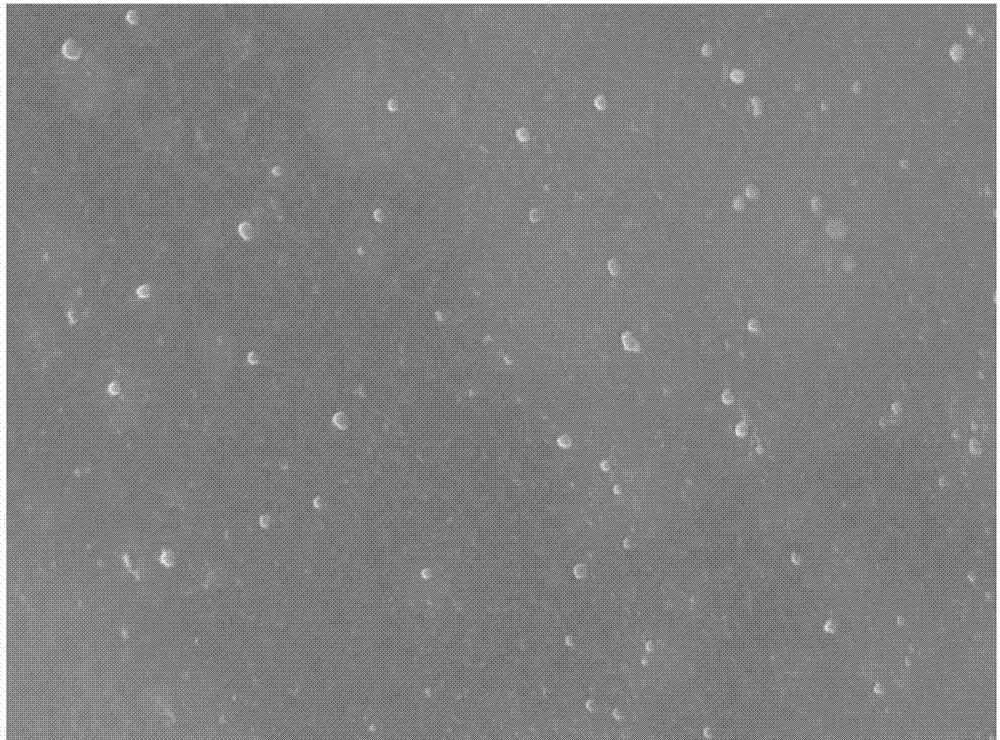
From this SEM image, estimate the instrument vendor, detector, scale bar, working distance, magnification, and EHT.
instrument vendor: JEOL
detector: SEI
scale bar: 1000 nm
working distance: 8.5 mm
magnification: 10 K X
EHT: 10 kV